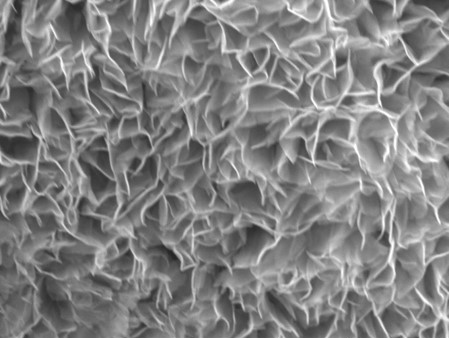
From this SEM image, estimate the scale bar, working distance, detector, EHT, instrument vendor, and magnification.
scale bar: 1000 nm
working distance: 9.1 mm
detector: SEI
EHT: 15 kV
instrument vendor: JEOL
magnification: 20 K X